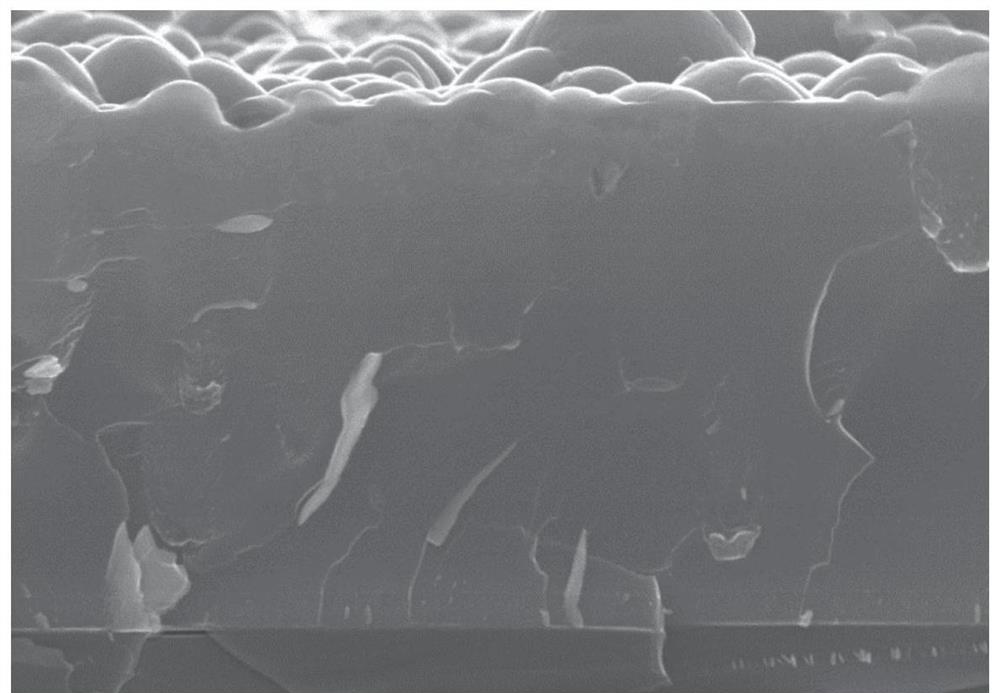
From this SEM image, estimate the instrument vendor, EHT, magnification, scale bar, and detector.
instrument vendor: Hitachi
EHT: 20 kV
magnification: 20 K X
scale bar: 2000 nm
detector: SE(U)